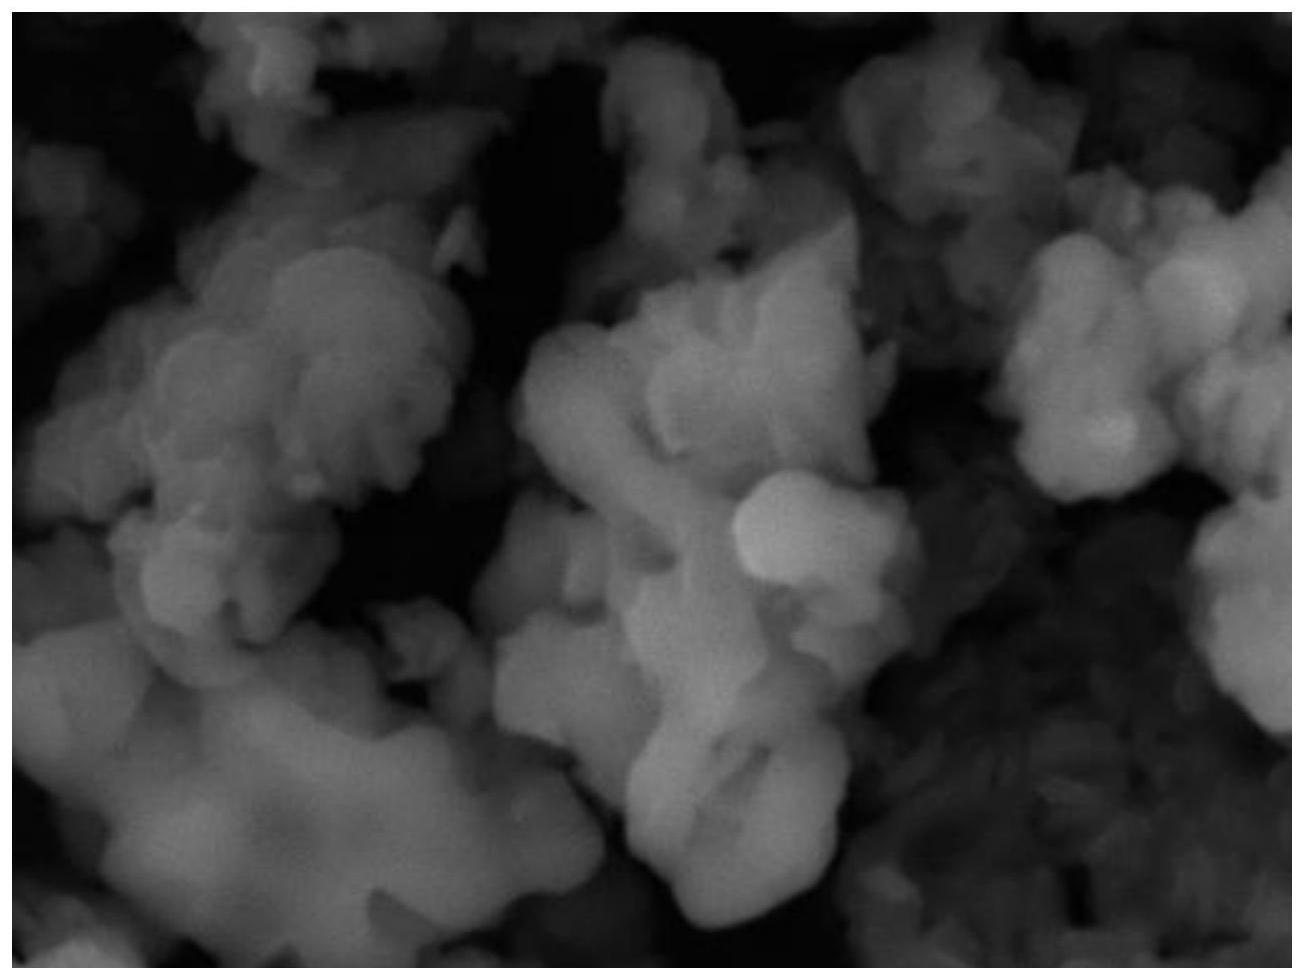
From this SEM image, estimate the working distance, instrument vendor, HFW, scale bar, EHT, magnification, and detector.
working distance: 13.69 mm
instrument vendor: Tescan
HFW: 12.8 µm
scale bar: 2000 nm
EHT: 30 kV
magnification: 10.8 K X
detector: SE